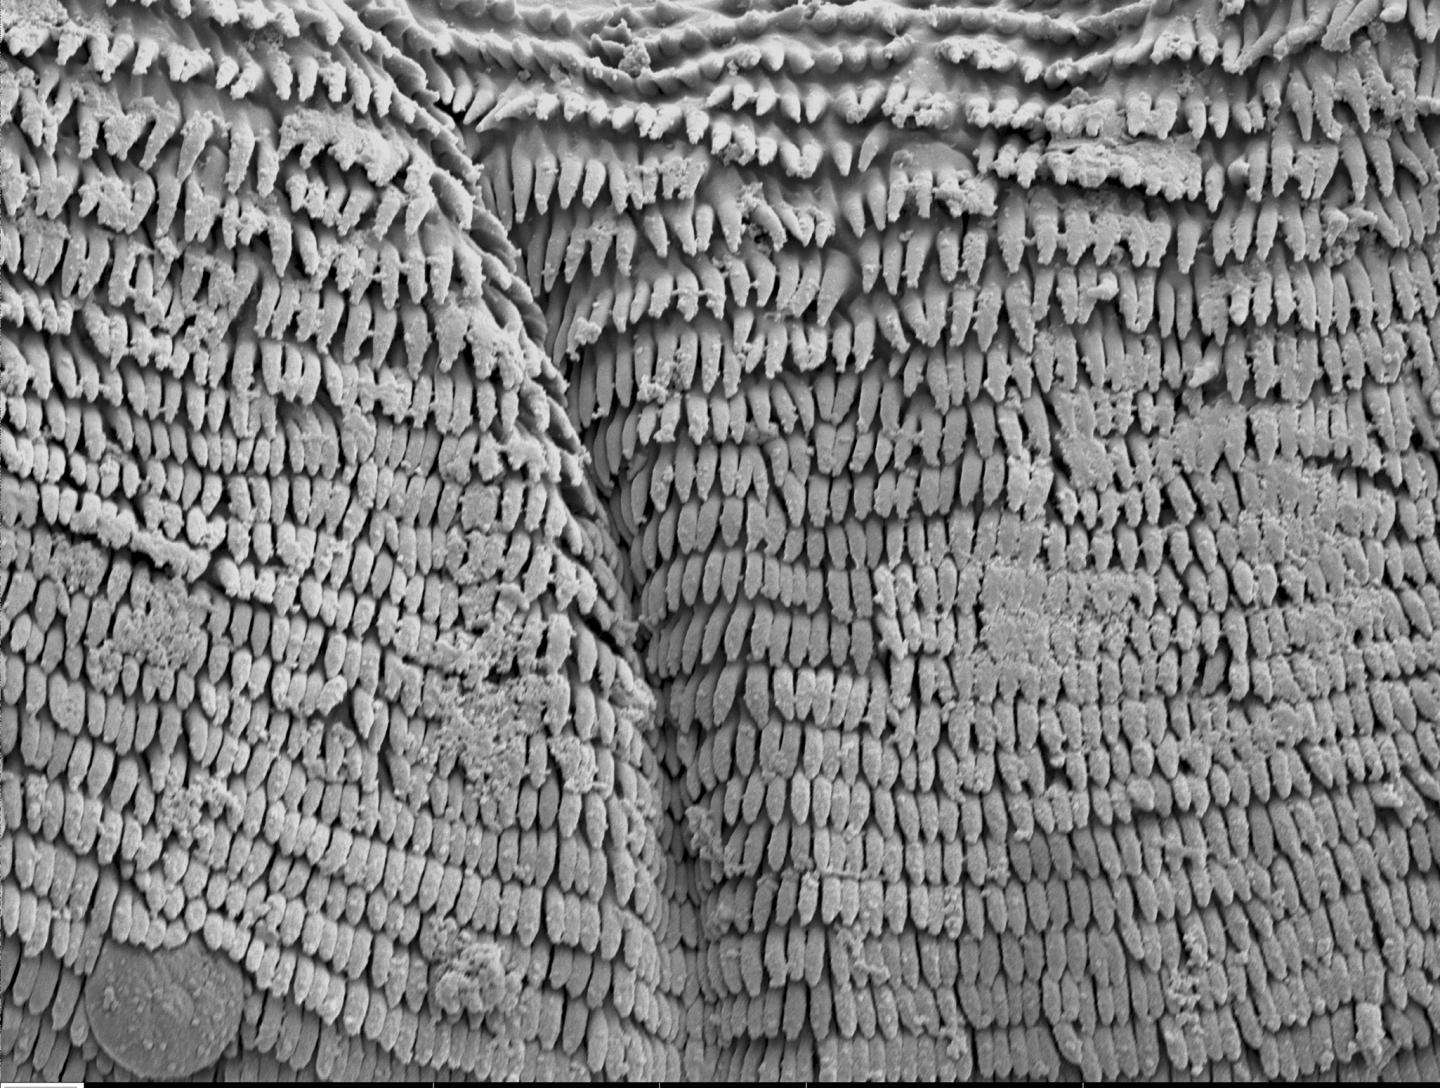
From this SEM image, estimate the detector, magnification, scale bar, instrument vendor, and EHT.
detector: SE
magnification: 3.5 K X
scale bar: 20000 nm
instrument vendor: other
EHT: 5 kV